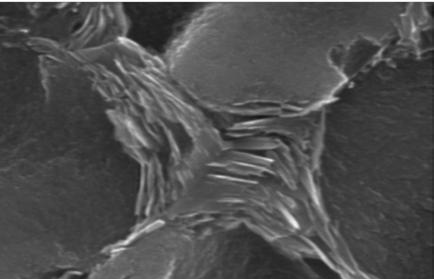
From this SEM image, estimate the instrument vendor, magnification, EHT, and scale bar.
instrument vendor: other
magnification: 15 K X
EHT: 20 kV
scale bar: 667 nm